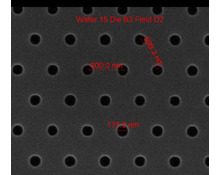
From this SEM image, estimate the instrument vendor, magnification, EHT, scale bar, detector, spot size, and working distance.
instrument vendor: FEI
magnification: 75 K X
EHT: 10 kV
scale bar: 1000 nm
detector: ETD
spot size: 1.5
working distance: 10.3 mm